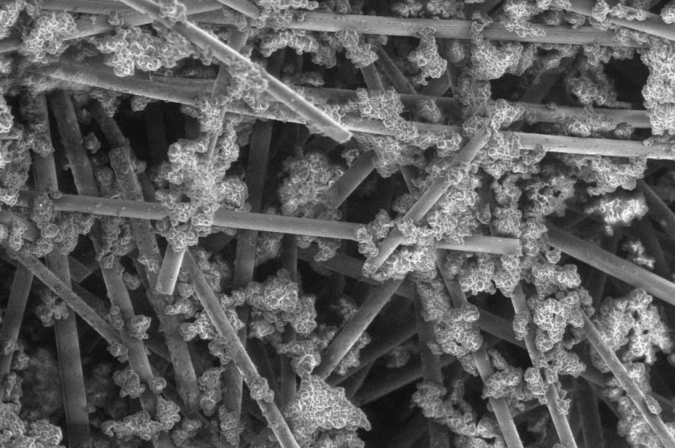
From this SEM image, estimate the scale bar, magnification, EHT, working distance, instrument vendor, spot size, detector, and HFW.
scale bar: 100000 nm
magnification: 1 K X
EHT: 5 kV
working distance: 9.2 mm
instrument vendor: FEI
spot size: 2.2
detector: LFD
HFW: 414 µm